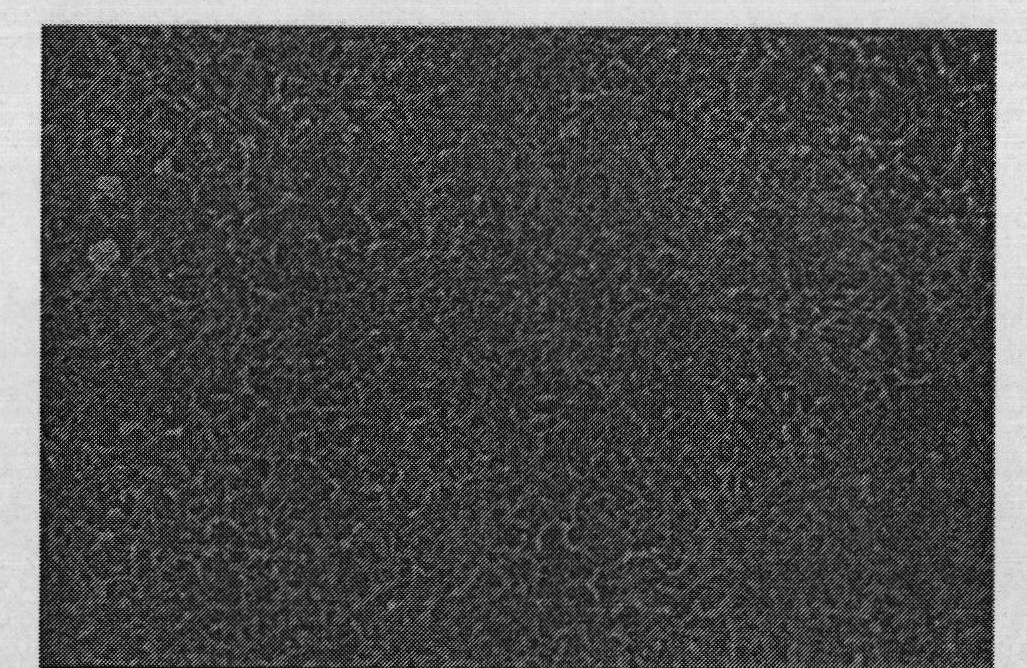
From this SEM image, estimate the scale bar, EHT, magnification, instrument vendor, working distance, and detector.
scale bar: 1000 nm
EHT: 10 kV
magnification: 50 K X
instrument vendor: Hitachi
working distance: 8.7 mm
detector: SE(M)